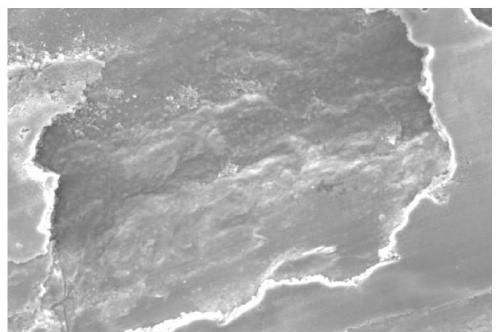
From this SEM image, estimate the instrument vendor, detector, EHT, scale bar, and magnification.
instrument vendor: JEOL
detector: SEI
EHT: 25 kV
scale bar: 50000 nm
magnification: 0.5 K X